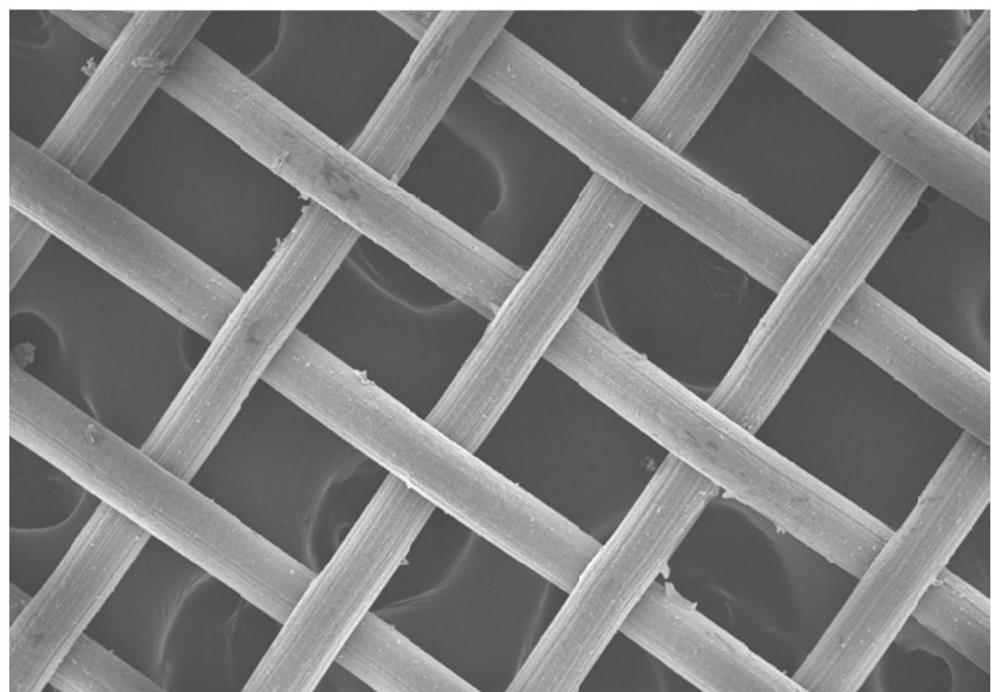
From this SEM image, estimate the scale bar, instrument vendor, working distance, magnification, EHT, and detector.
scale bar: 100000 nm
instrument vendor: Zeiss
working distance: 6.4 mm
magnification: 0.1 K X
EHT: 10 kV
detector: InLens